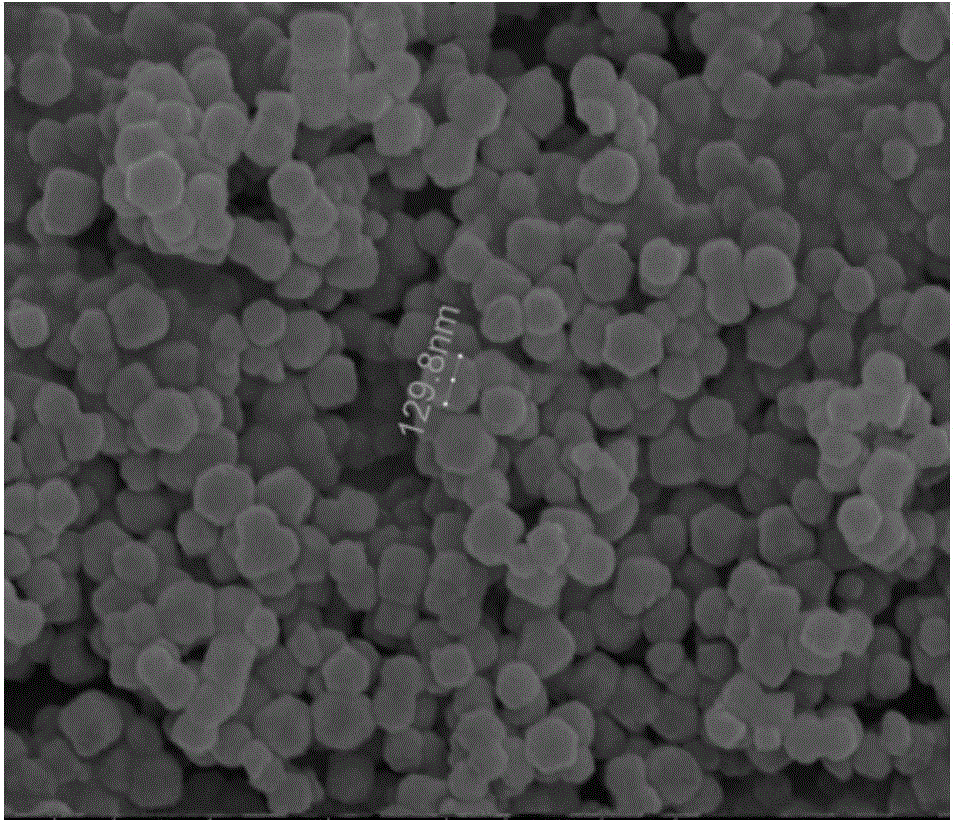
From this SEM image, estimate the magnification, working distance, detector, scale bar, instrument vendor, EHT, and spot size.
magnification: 100 K X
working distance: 5 mm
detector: TLD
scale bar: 500 nm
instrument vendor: FEI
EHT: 10 kV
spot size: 3